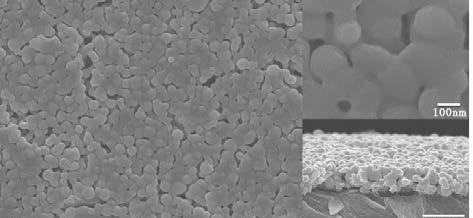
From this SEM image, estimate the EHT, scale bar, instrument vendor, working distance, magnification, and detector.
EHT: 10 kV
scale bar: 1000 nm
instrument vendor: JEOL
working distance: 8.1 mm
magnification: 20 K X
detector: SEI